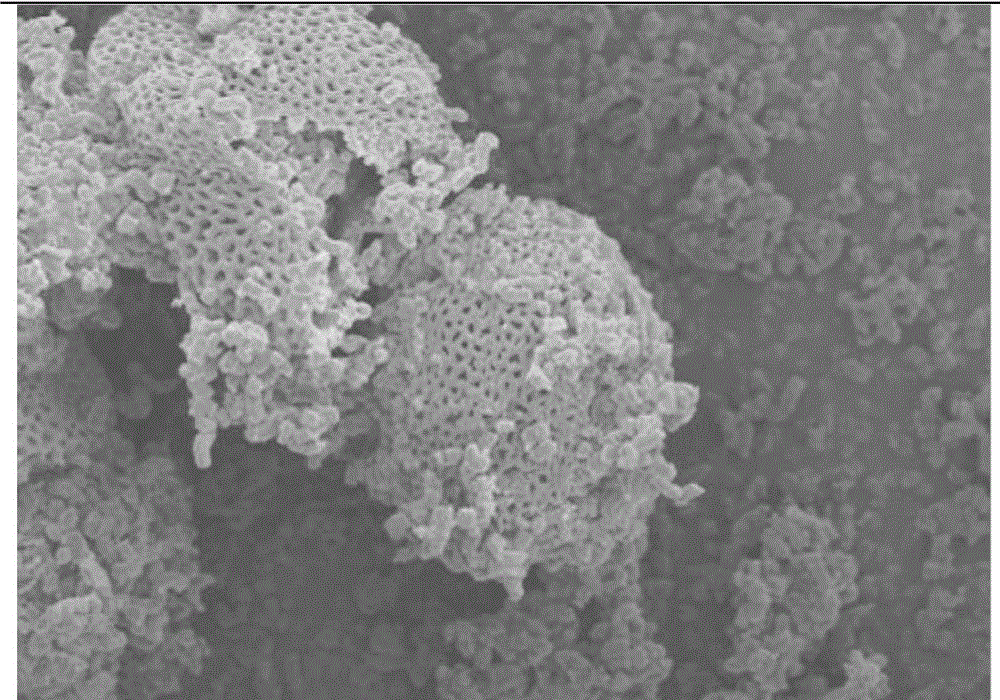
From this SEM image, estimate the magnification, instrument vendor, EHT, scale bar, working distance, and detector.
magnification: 2 K X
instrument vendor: Hitachi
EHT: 5 kV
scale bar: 20000 nm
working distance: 9.1 mm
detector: SE(M)